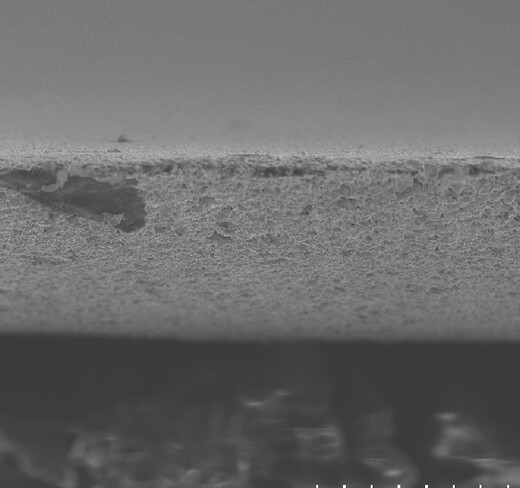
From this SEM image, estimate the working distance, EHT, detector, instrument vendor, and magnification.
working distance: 9.9 mm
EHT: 10 kV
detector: SE(UL)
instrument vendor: Hitachi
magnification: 1 K X